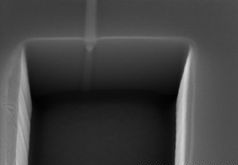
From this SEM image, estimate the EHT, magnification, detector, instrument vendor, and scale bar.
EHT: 10 kV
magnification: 50 K X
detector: SE(M)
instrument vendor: Hitachi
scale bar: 1000 nm